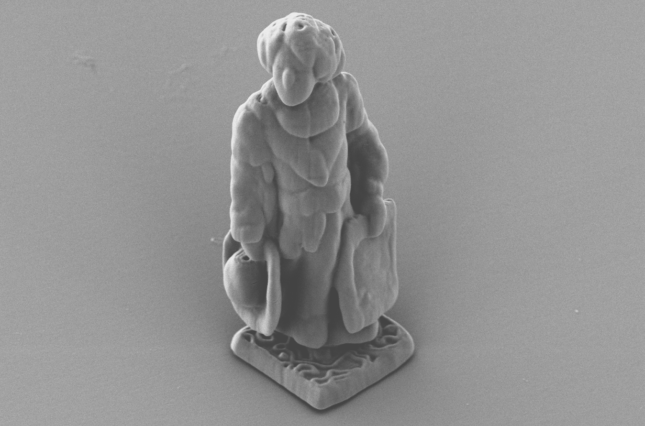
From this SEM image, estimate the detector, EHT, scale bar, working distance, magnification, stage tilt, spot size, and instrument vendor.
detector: T1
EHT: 3 kV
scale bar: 10000 nm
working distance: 12.2346 mm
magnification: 10 K X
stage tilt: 0°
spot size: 7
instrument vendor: FEI/Thermo